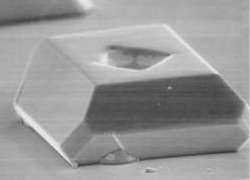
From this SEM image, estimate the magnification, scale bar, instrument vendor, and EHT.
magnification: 0.5 K X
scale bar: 100000 nm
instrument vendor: JEOL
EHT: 30 kV